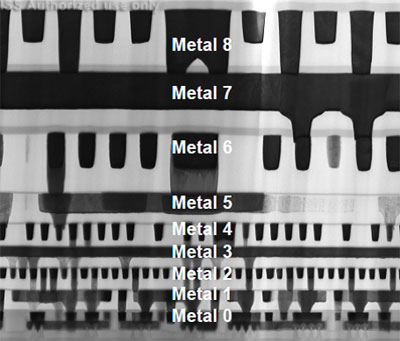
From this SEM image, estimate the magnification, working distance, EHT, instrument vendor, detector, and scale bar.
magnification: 80 K X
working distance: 4.1 mm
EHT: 30 kV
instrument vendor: FEI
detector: STEM II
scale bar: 1000 nm